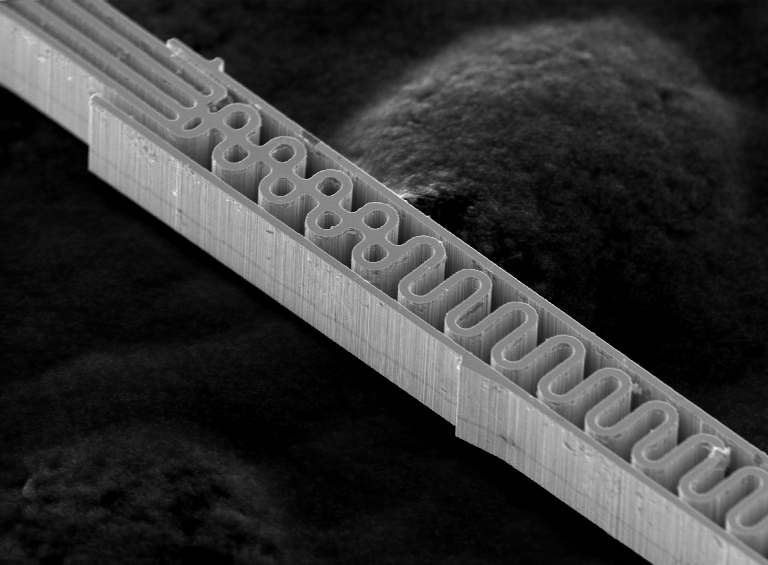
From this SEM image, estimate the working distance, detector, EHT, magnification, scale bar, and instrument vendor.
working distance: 9.9 mm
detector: SEI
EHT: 20 kV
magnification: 0.6 K X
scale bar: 50000 nm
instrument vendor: JEOL